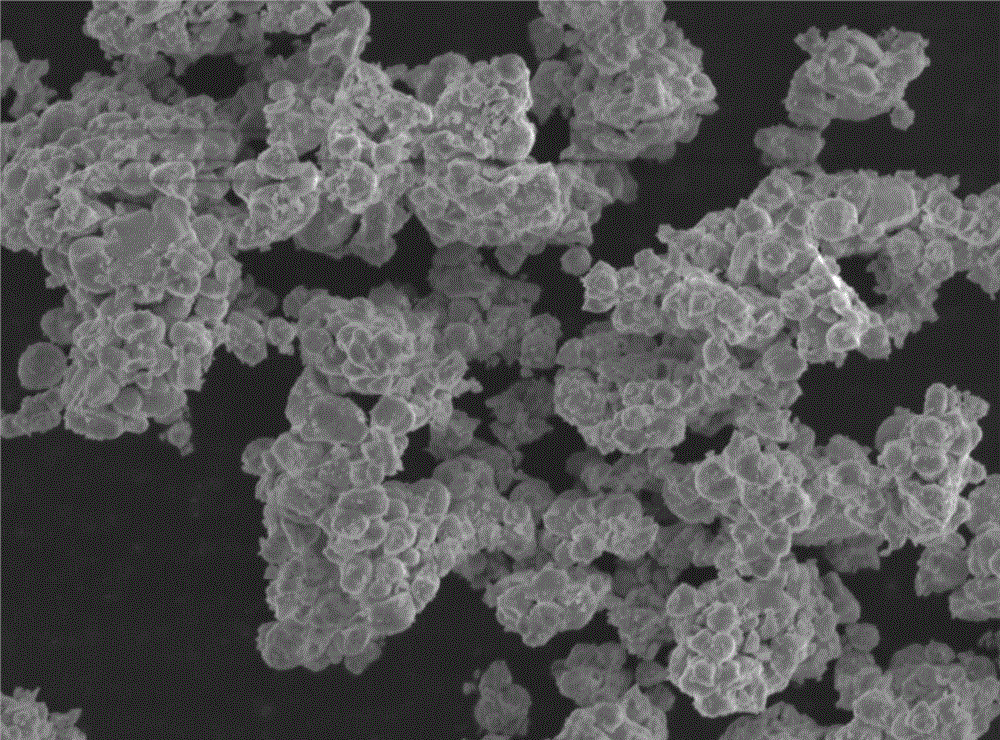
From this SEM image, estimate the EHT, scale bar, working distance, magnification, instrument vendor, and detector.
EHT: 8 kV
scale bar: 1000 nm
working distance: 9.7 mm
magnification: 10 K X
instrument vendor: JEOL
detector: LED